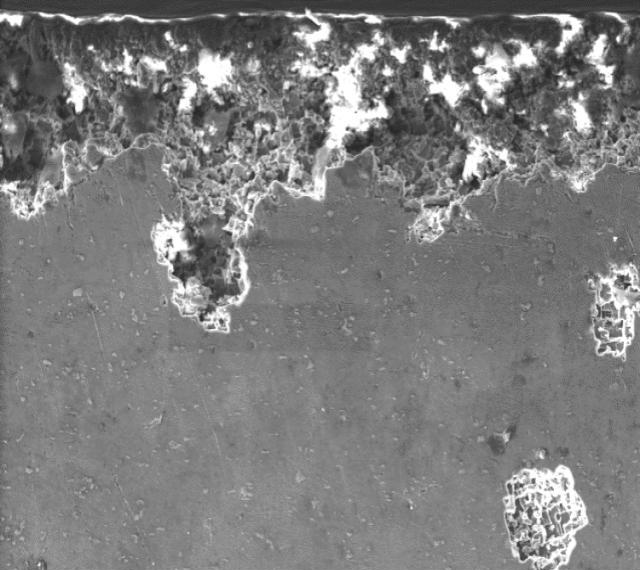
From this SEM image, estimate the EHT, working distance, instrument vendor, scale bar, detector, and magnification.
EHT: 16 kV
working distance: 11.5 mm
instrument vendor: Zeiss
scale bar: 20000 nm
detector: SE1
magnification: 0.5 K X